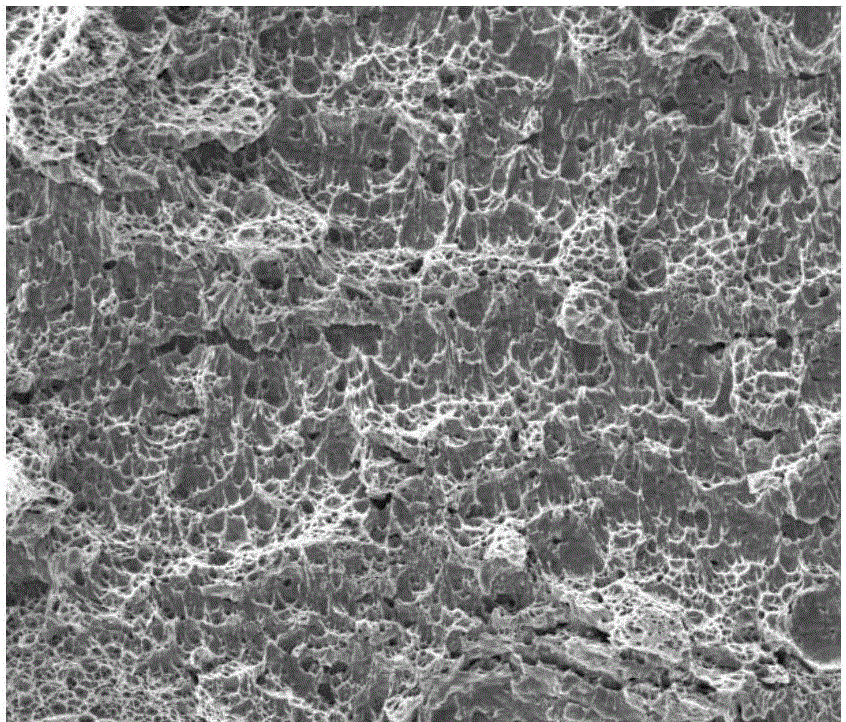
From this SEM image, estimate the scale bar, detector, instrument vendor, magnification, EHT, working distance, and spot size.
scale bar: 100000 nm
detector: ETD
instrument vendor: FEI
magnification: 0.5 K X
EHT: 20 kV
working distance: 11.8 mm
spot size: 4.5